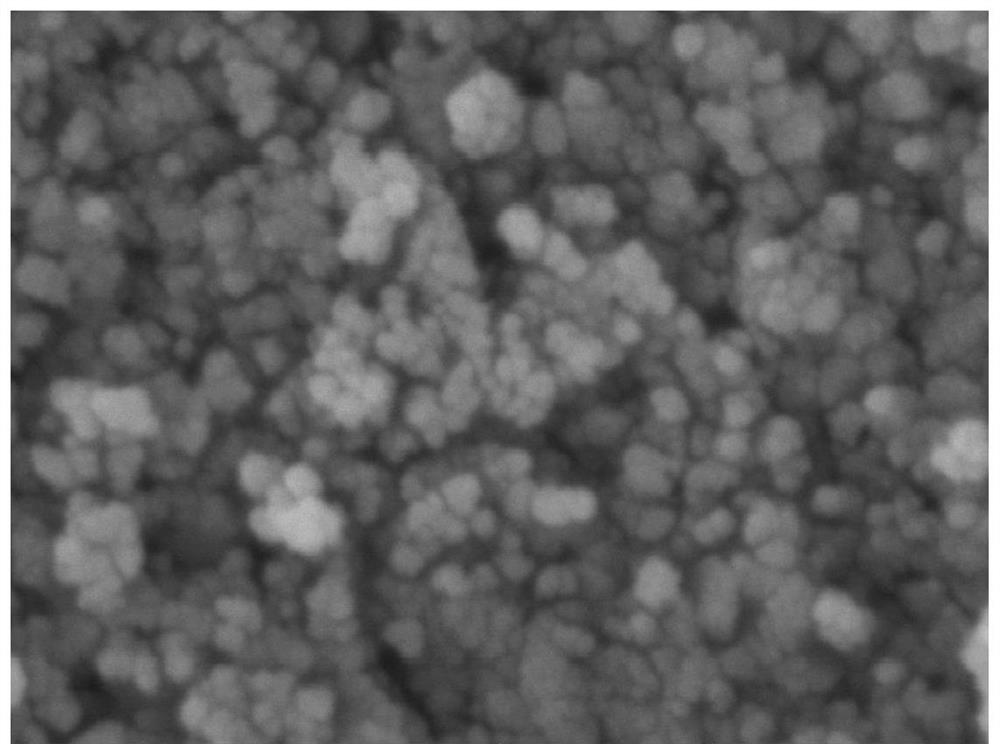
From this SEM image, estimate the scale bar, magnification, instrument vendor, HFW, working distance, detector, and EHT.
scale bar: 200 nm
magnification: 300 K X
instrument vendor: Tescan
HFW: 0.923 µm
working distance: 5.02 mm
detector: In-Beam SE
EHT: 5 kV